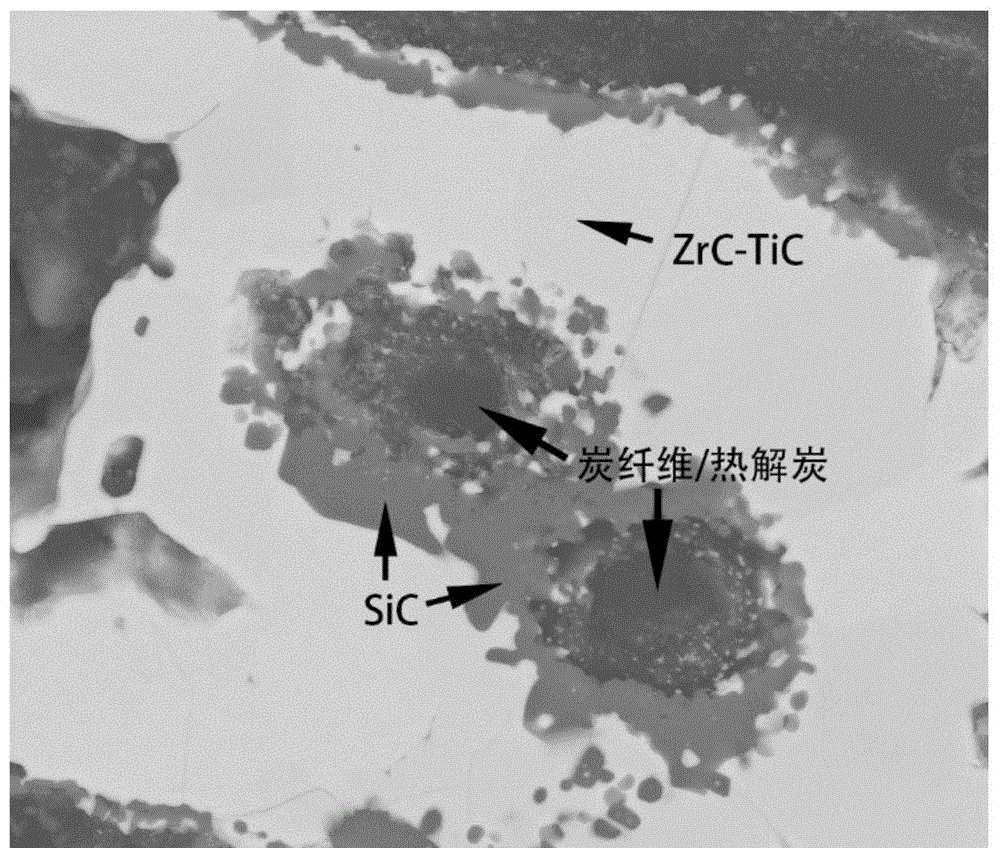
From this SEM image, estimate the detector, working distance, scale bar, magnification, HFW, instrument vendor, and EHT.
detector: BSED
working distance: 10.9 mm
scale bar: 20000 nm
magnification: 5 K X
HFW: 59.7 µm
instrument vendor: FEI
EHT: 20 kV